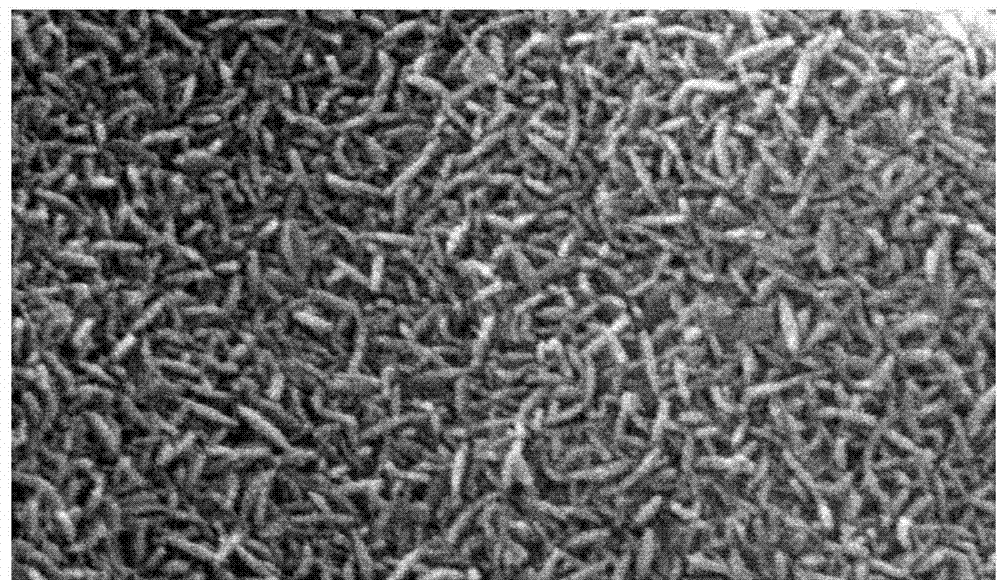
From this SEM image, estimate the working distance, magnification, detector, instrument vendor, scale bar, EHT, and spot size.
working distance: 4.3 mm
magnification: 40 K X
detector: SE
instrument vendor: FEI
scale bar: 500 nm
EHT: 20 kV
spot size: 1